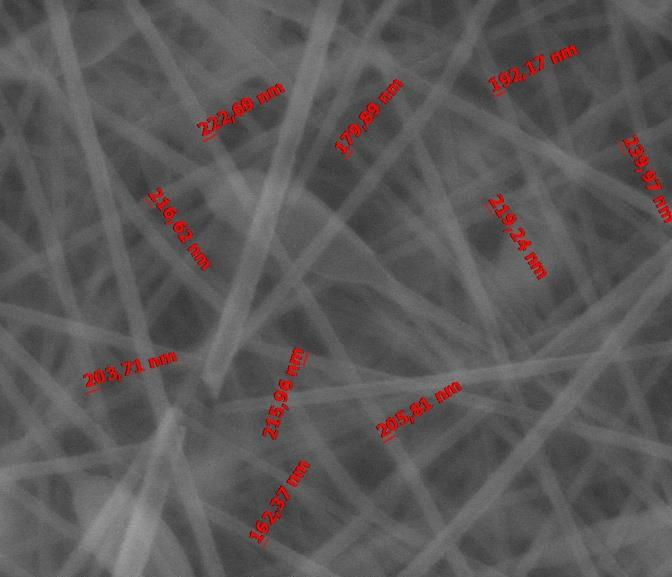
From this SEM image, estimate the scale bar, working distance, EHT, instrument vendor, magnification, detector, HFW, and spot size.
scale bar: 3000 nm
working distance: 5.2 mm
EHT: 18 kV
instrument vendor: FEI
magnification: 30 K X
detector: LVD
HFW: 9.95 µm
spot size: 4.5